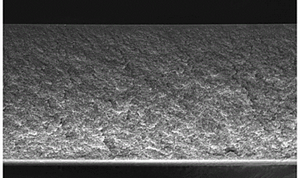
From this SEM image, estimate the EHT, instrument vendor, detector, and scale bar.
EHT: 10 kV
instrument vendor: Zeiss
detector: InLens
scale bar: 10000 nm